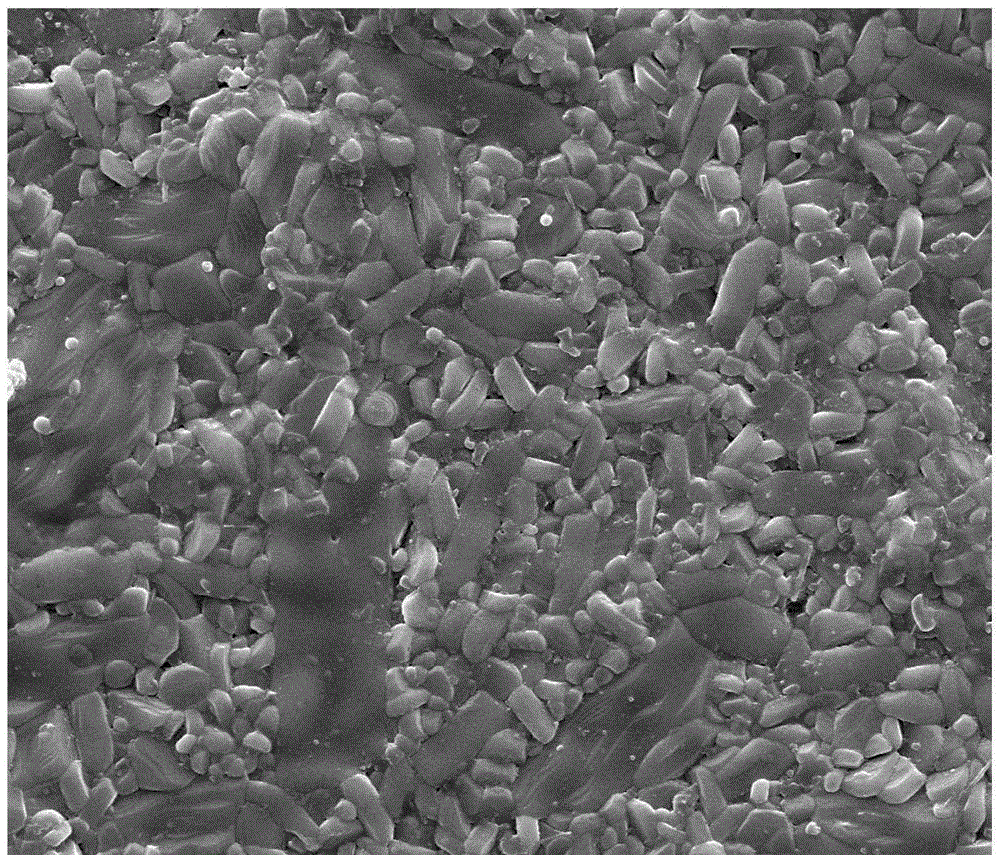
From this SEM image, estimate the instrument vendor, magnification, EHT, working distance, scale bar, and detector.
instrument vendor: FEI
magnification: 10 K X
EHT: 20 kV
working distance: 11.3 mm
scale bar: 10000 nm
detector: SE